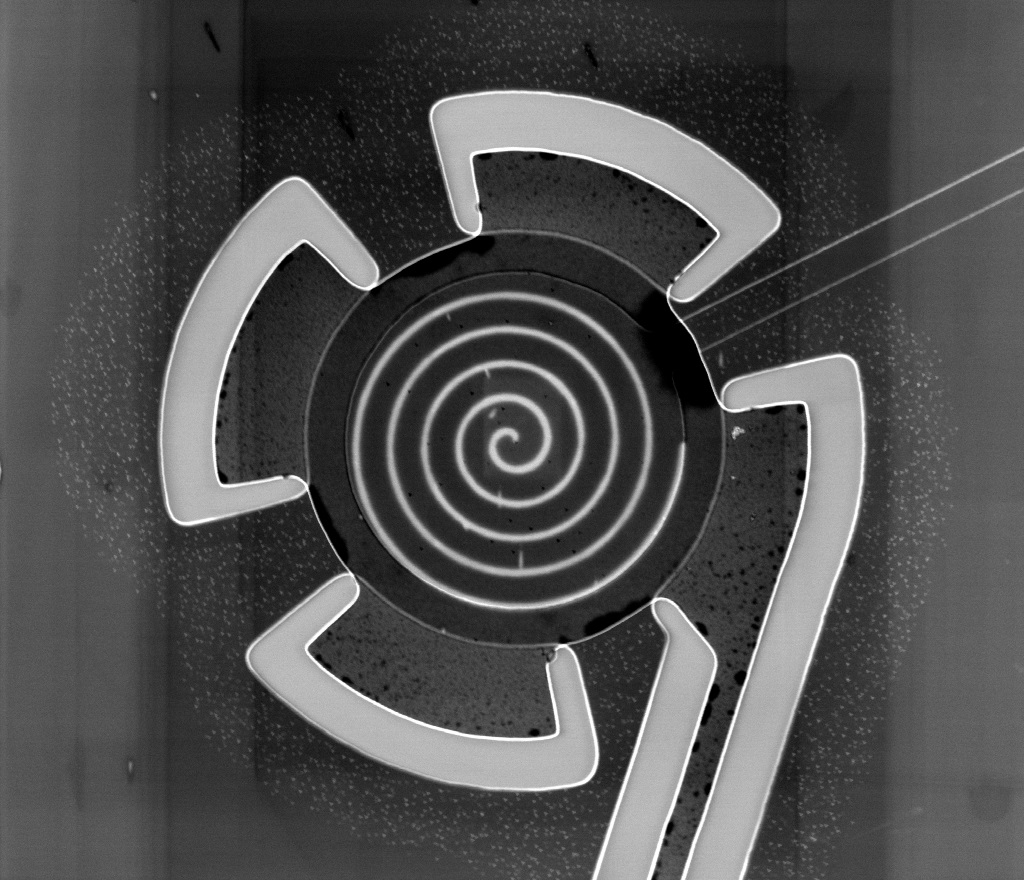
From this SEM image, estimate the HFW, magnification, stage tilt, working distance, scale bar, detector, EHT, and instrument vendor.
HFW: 73.1 µm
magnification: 3.5 K X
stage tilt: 0°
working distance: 4.9 mm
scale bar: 30000 nm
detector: TLD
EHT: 2 kV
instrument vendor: FEI